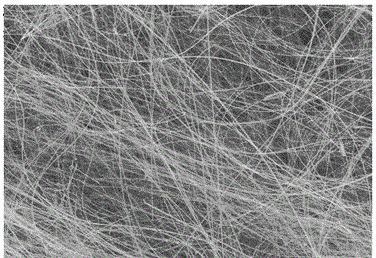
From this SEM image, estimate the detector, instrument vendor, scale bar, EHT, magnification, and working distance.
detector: SE(M)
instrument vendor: Hitachi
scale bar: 200000 nm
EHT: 15 kV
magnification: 0.2 K X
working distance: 11.4 mm